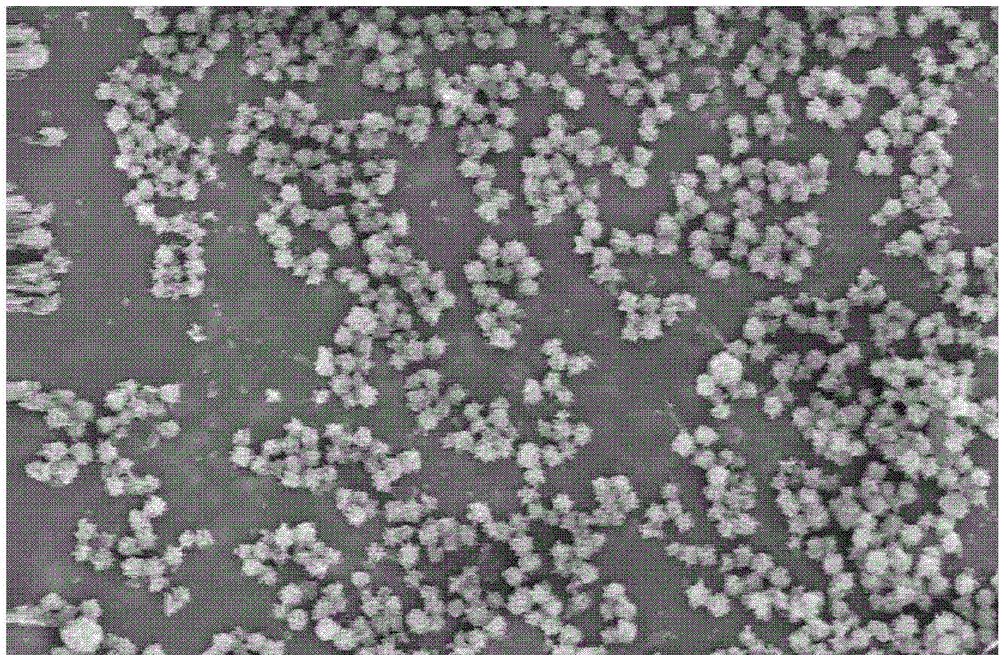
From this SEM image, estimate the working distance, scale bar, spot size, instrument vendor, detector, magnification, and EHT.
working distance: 6.7 mm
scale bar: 2000 nm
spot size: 4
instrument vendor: FEI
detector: TLD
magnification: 20 K X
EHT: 5 kV